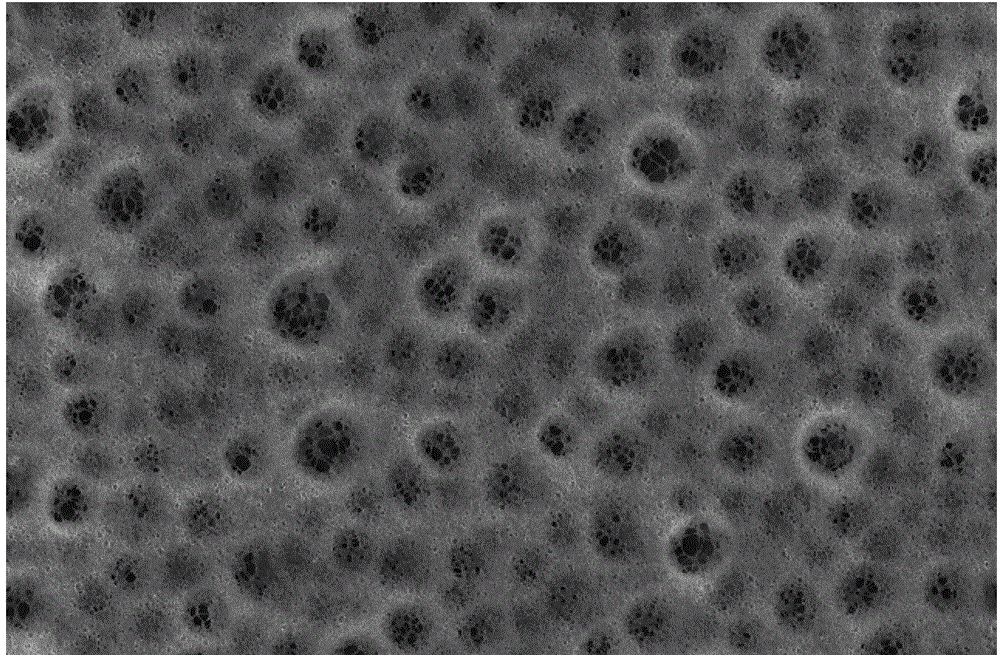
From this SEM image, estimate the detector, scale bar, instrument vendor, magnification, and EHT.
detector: SEI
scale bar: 50000 nm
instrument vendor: JEOL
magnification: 0.5 K X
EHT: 20 kV